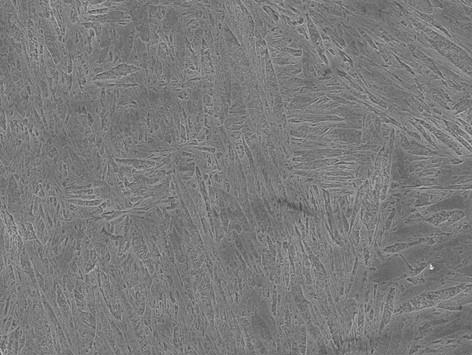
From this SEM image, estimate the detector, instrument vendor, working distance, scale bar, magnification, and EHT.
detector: SEI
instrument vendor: JEOL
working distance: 10 mm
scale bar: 1000 nm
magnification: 5 K X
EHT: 15 kV